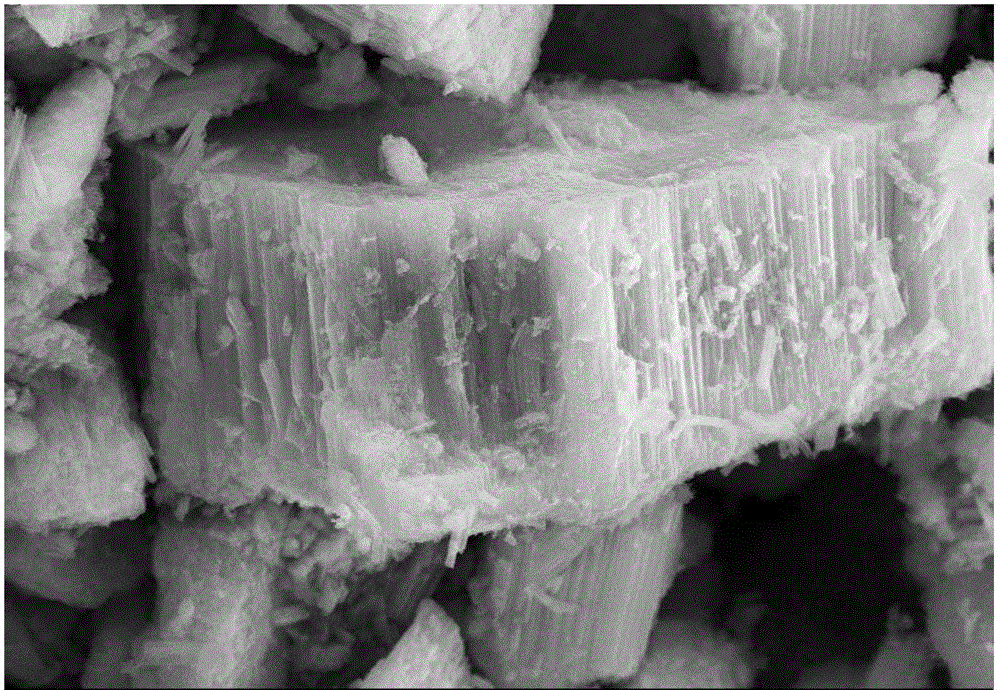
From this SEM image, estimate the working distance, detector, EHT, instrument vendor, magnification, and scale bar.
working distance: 9.5 mm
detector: SE2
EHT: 15 kV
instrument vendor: Zeiss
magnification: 17.84 K X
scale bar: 2000 nm